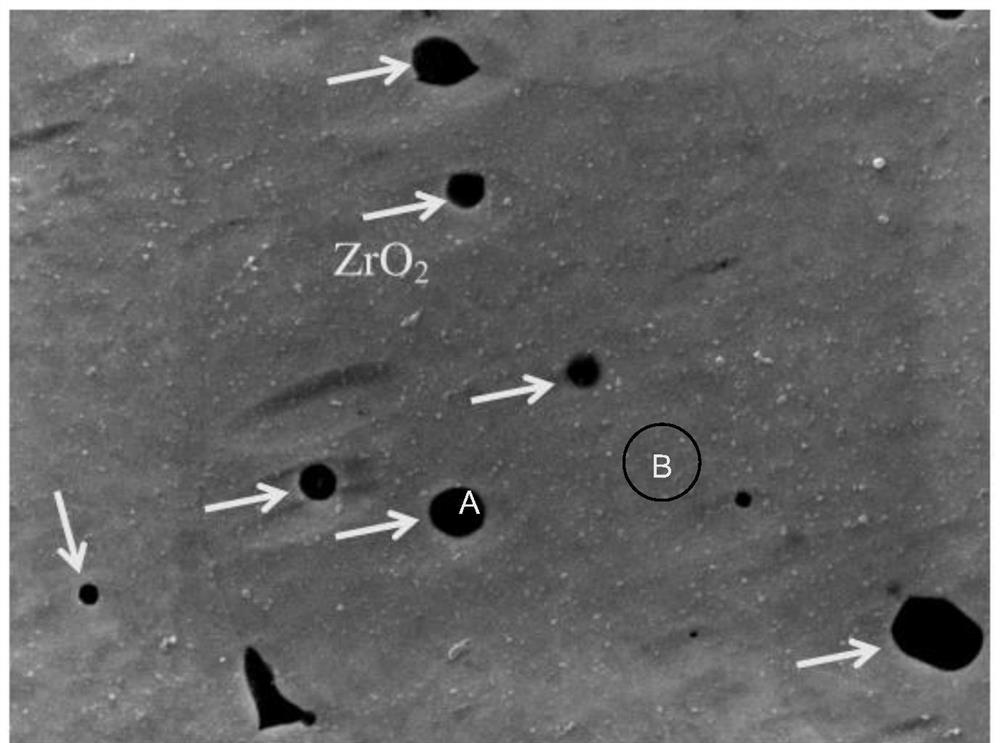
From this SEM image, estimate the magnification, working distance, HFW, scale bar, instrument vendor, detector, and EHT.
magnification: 11 K X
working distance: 11.1 mm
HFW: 11.6 µm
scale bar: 1000 nm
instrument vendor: JEOL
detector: SED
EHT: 10 kV